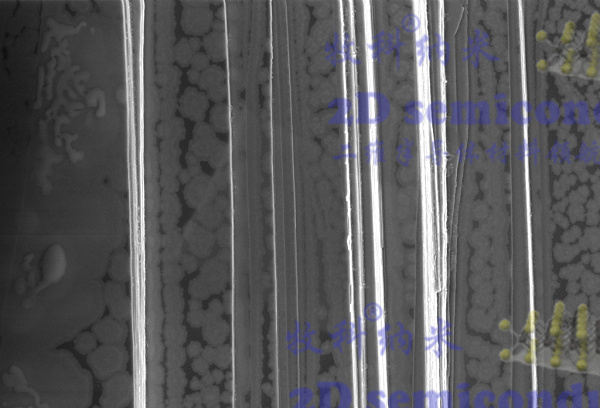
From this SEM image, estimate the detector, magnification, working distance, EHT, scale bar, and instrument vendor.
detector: InLens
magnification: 10 K X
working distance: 6.3 mm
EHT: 3 kV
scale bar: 1000 nm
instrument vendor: Zeiss